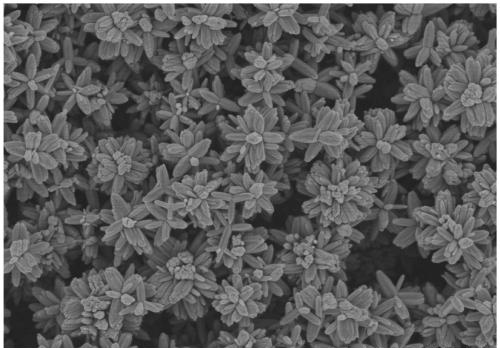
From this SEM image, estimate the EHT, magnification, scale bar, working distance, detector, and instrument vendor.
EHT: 3 kV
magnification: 20 K X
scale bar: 2000 nm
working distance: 7.7 mm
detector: SE(U)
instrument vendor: Hitachi